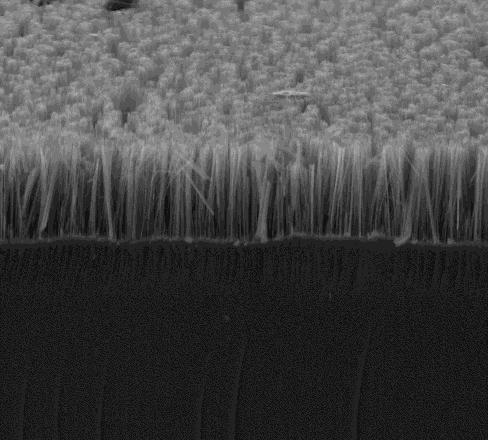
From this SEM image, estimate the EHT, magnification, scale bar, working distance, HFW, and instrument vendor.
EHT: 10 kV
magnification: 5 K X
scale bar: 30000 nm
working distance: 4.9 mm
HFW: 59.7 µm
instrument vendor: FEI